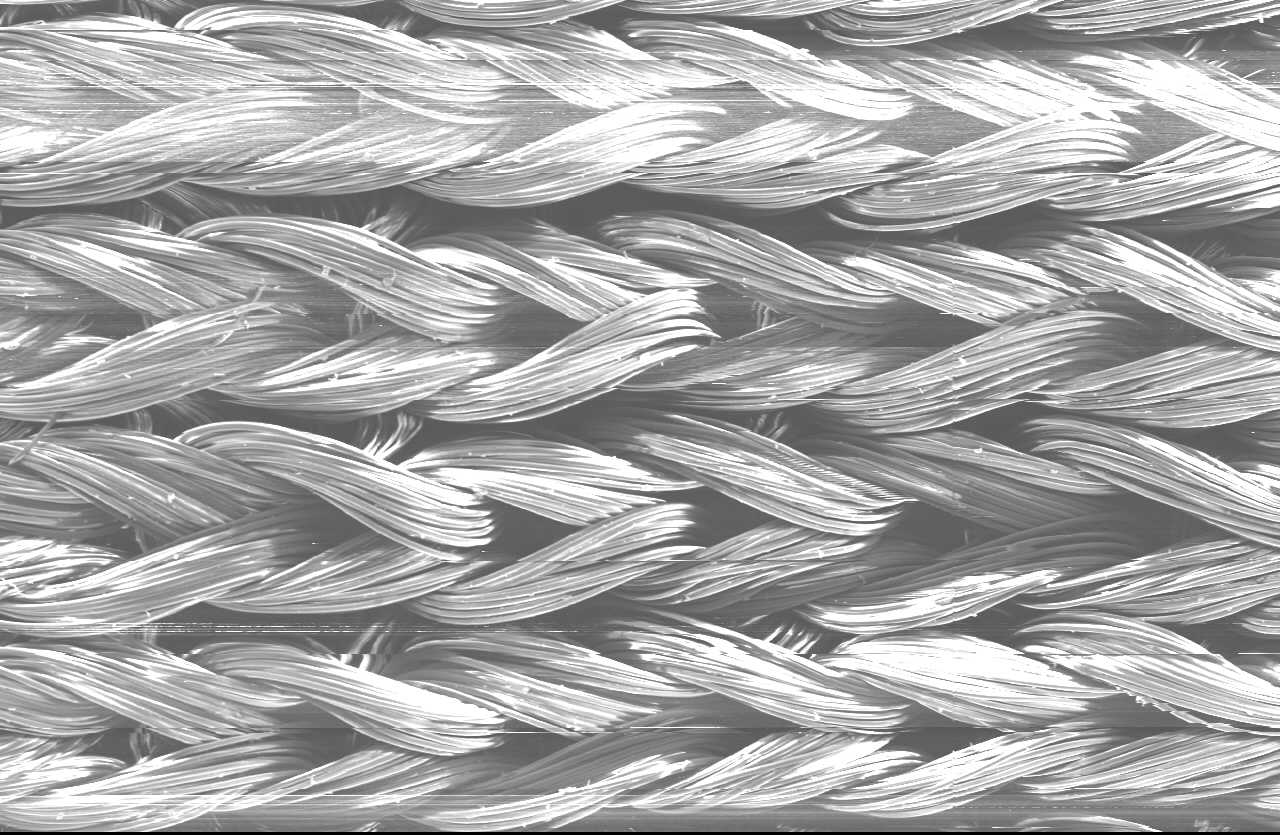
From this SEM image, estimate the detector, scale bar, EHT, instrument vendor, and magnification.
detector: SEI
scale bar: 500000 nm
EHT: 15 kV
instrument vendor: JEOL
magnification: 0.035 K X